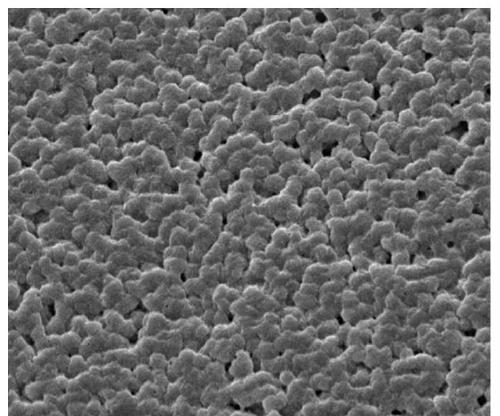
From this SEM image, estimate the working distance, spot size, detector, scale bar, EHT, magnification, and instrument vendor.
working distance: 11.4 mm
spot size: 5.5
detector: ETD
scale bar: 20000 nm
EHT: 12.5 kV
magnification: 2 K X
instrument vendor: FEI